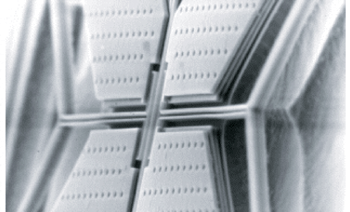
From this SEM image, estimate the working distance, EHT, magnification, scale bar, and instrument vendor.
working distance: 17 mm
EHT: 15 kV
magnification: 1.5 K X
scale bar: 10000 nm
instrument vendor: JEOL